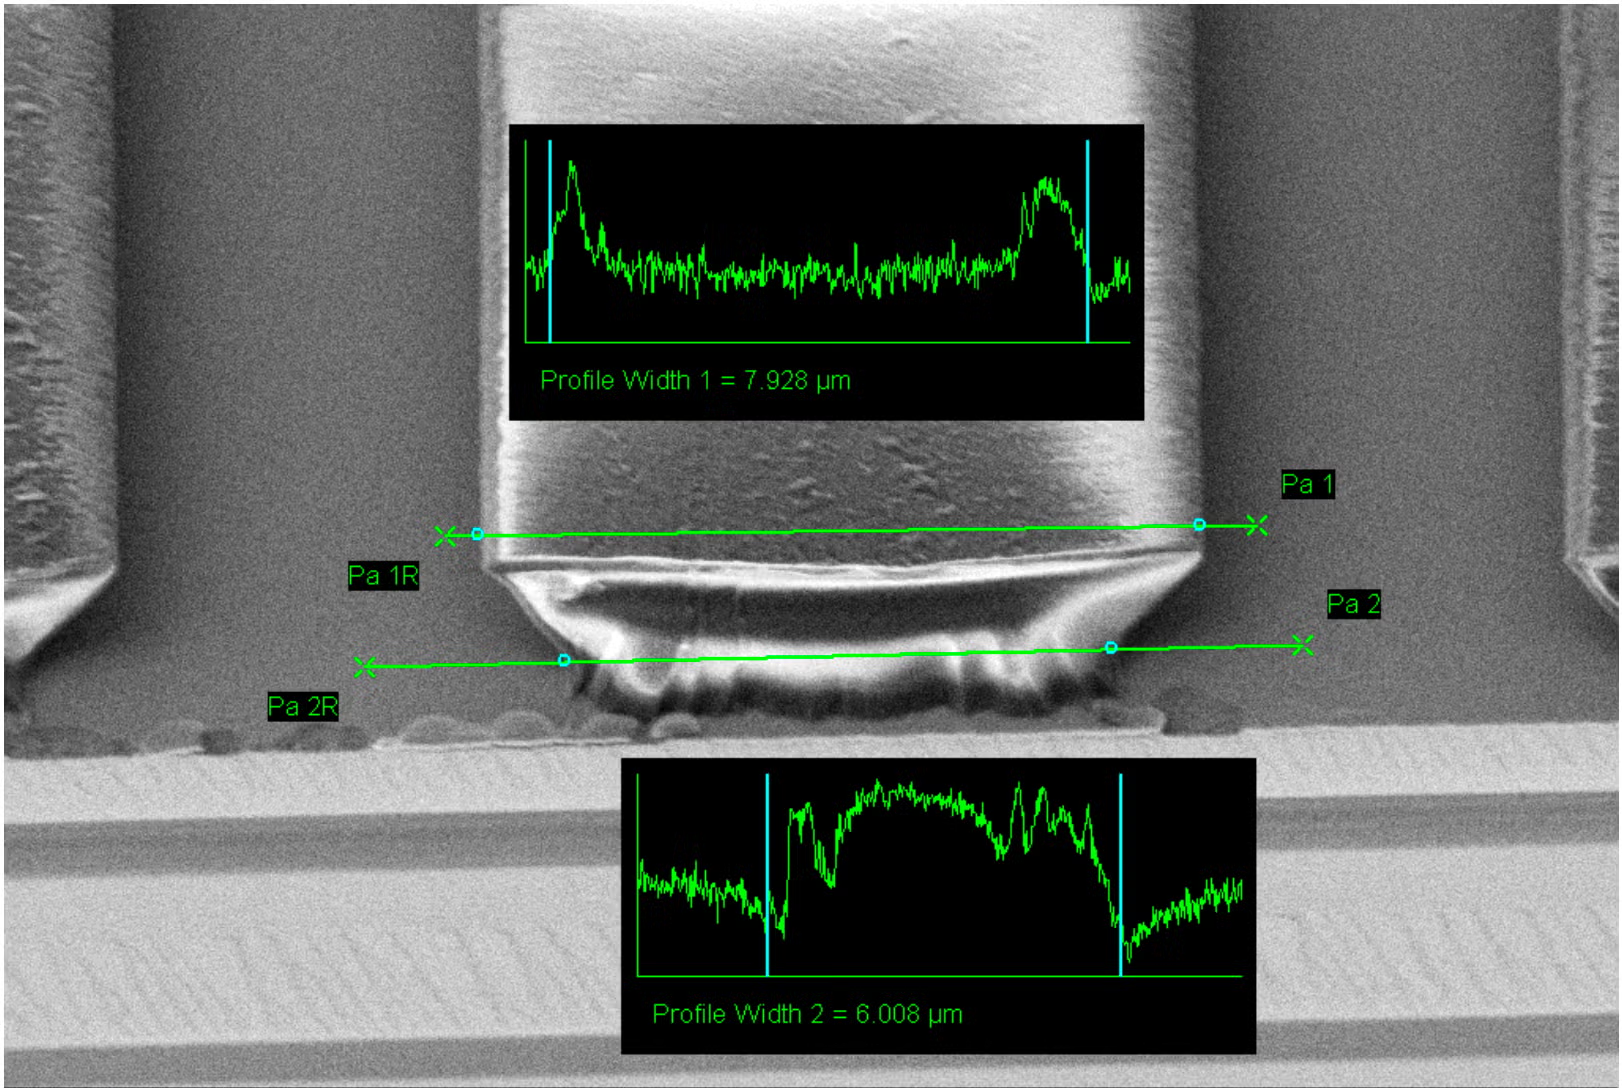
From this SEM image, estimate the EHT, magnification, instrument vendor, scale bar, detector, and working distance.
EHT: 2 kV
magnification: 6.45 K X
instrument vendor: Zeiss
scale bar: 2000 nm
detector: SE2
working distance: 8 mm